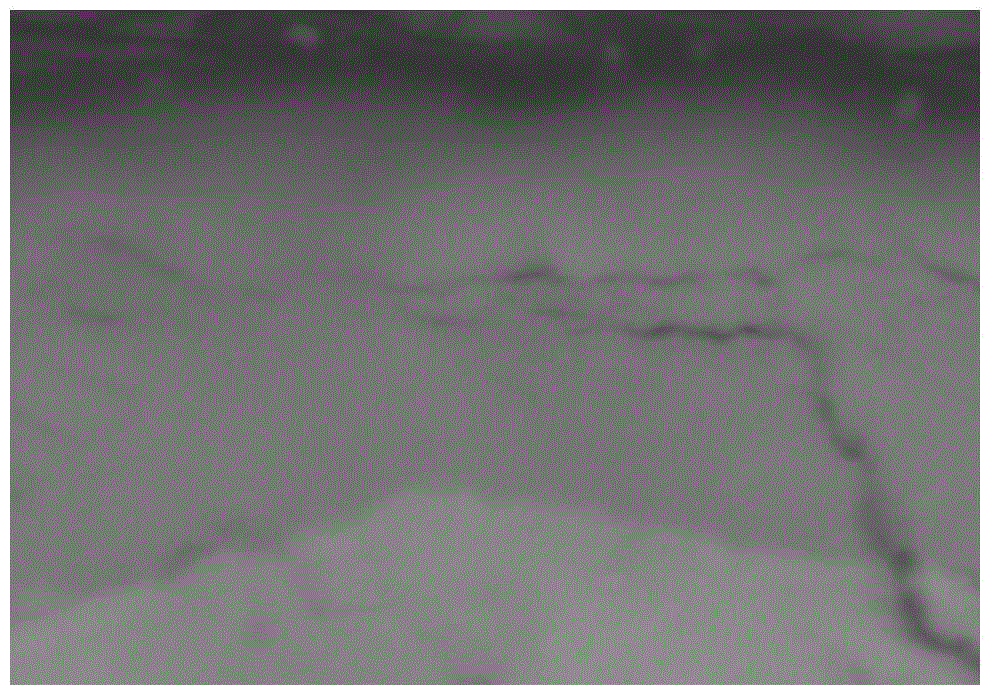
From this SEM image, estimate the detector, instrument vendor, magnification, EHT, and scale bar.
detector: BSECOMP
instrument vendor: Hitachi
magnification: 10 K X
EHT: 20 kV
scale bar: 5000 nm